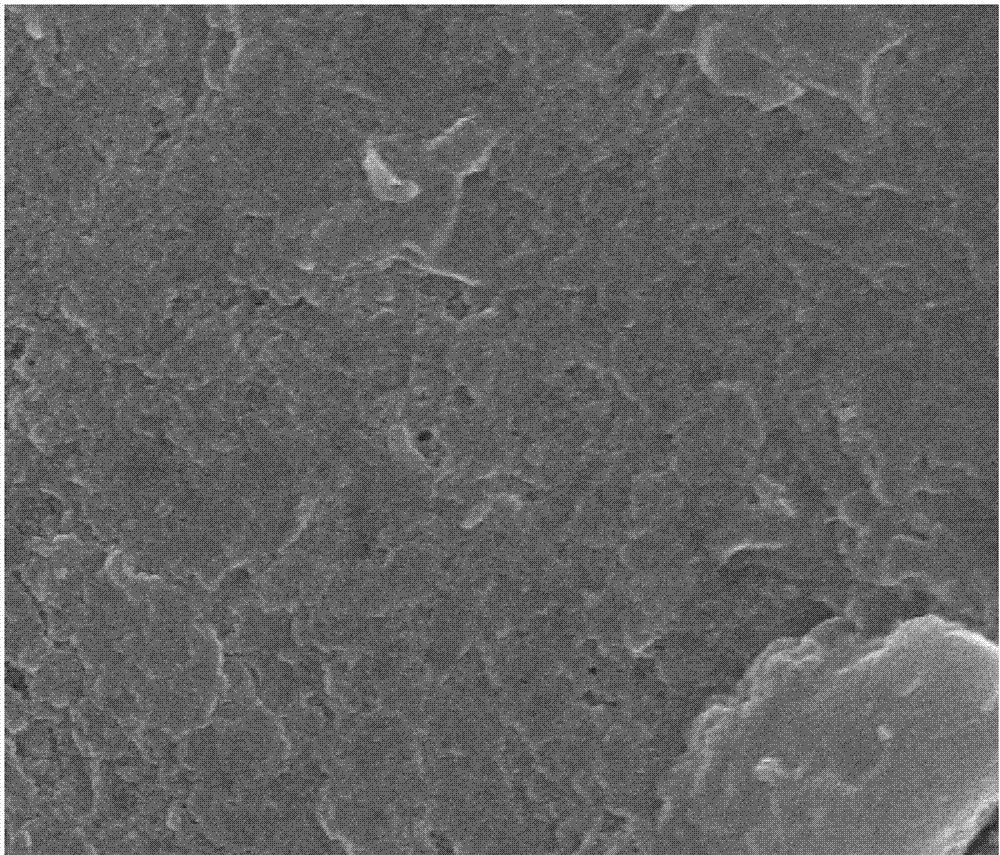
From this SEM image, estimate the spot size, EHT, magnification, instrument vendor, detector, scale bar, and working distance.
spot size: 3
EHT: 10 kV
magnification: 40 K X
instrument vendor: FEI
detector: TLD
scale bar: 1000 nm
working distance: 4.7 mm